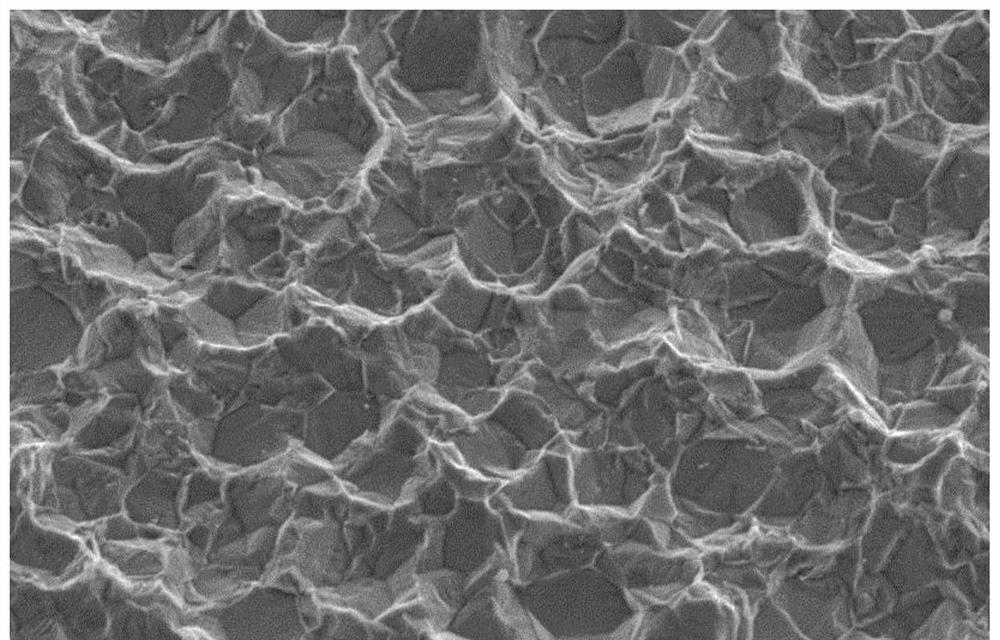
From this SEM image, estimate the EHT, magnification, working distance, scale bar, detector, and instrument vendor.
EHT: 10 kV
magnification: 3 K X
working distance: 10 mm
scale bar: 10000 nm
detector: SE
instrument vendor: Hitachi